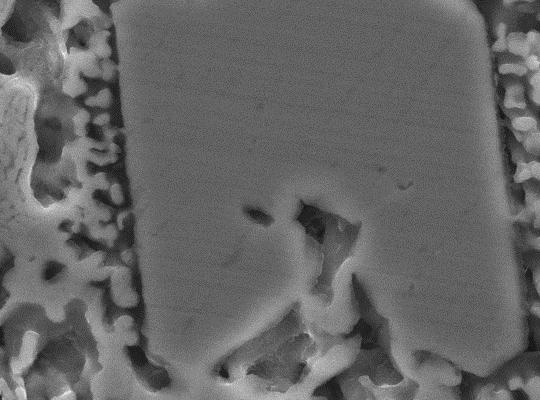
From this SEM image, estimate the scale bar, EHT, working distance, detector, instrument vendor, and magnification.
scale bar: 1000 nm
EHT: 15 kV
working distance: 10.2 mm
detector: SEI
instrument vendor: JEOL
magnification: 10 K X